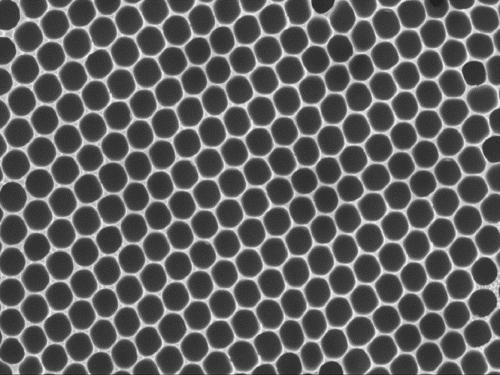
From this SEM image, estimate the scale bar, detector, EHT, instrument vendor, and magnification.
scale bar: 10000 nm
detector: SEI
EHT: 15 kV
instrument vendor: JEOL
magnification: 1 K X